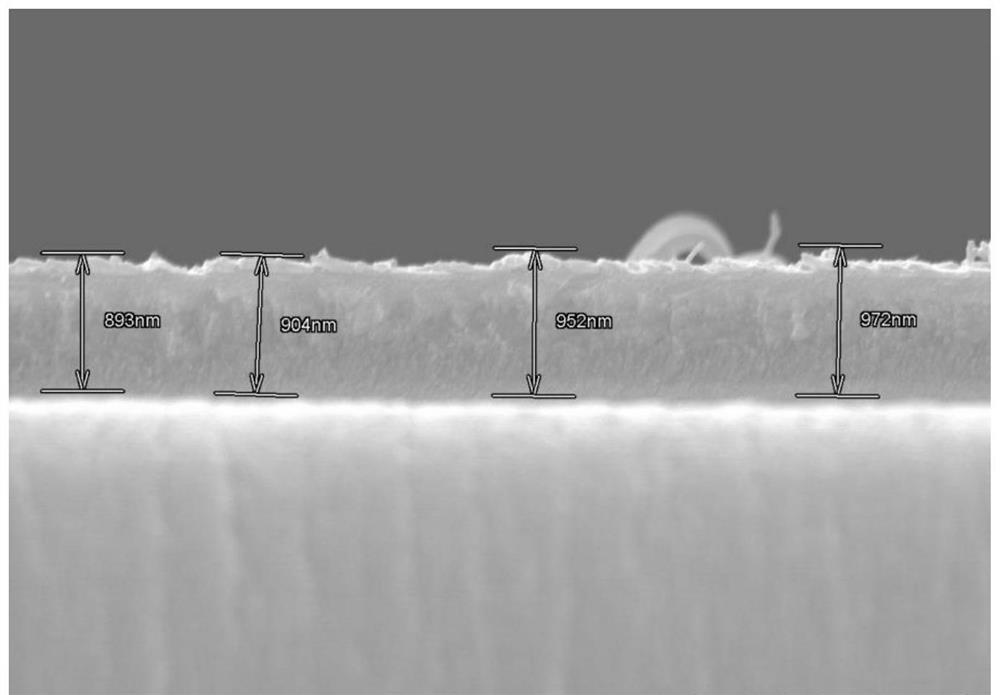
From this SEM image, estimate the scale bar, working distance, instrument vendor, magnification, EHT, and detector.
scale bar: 2000 nm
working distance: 8.5 mm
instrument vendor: Hitachi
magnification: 20 K X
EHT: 10 kV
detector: SE(M)